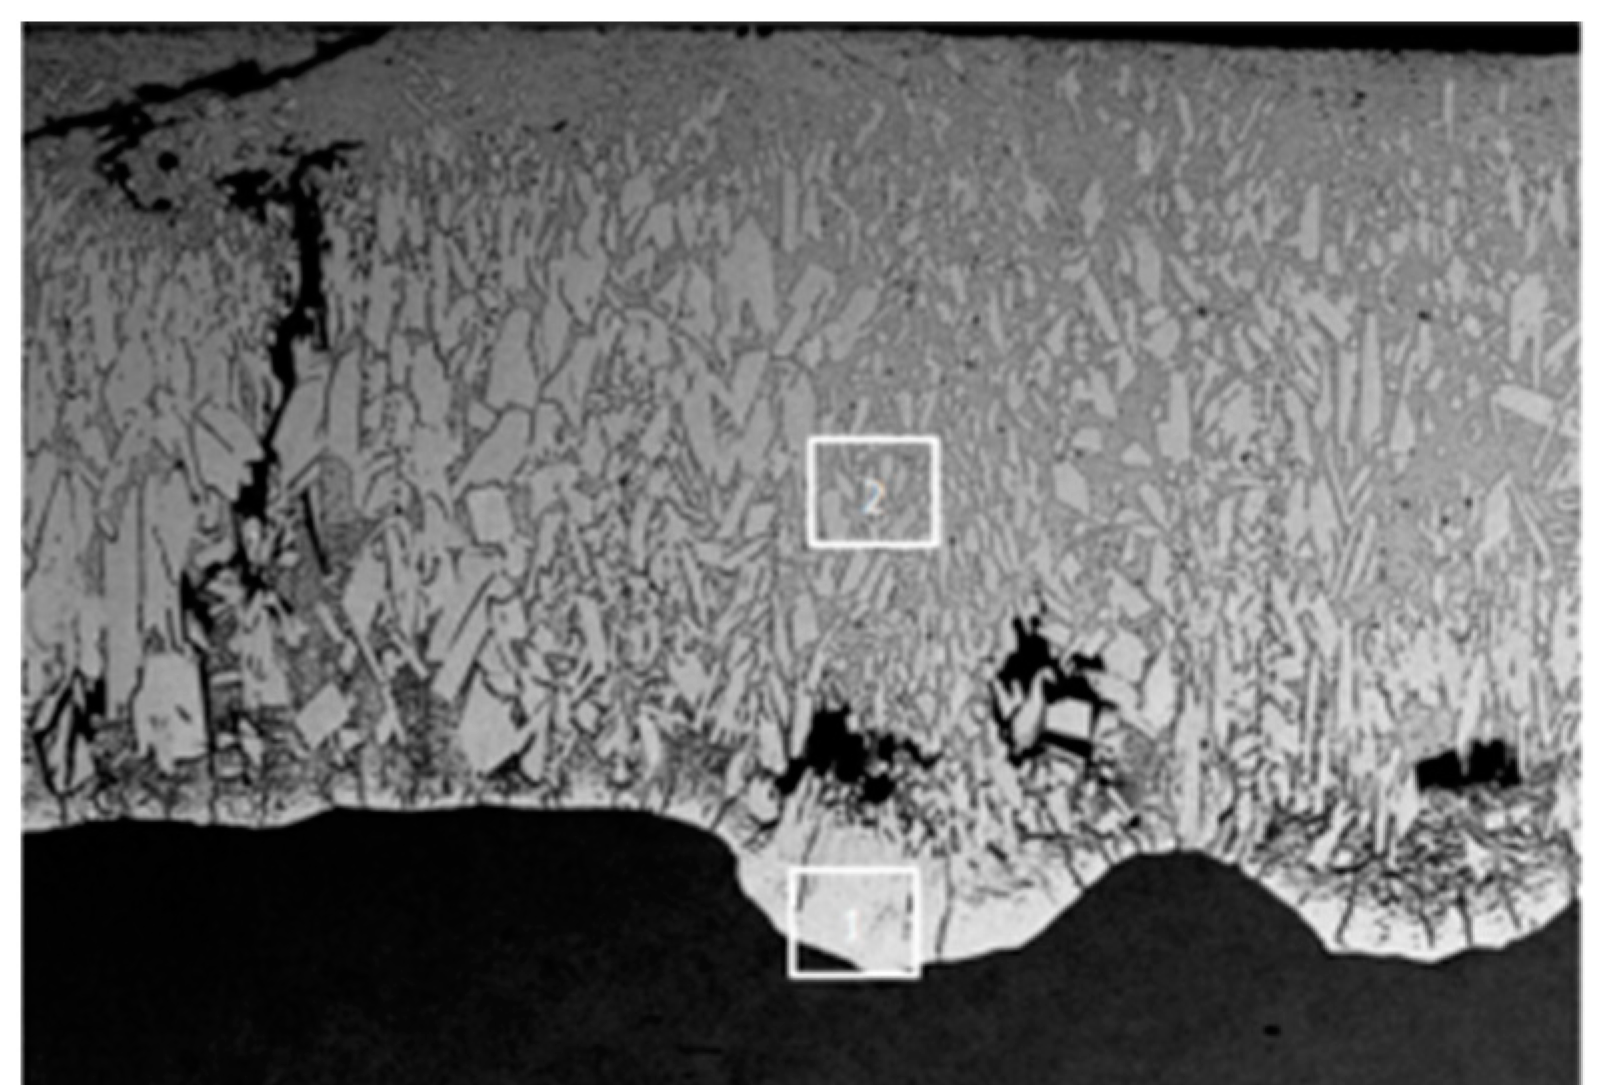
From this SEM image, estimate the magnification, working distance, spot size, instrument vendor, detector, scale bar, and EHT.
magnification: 0.3 K X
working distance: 10 mm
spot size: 60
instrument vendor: JEOL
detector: BEC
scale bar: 50000 nm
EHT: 15 kV